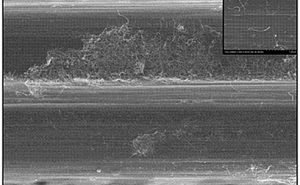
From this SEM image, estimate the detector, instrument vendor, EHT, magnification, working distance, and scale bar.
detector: SE(M)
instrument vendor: Hitachi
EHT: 5 kV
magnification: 7 K X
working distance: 6.6 mm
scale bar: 5000 nm